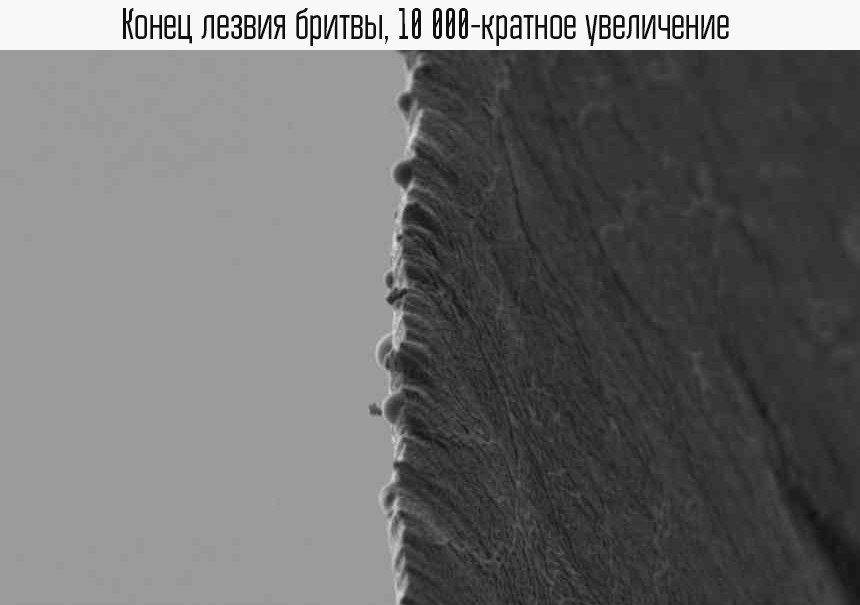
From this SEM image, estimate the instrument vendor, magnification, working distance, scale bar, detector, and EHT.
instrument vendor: Zeiss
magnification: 10 K X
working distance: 6.1 mm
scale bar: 1000 nm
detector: SE2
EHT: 1 kV